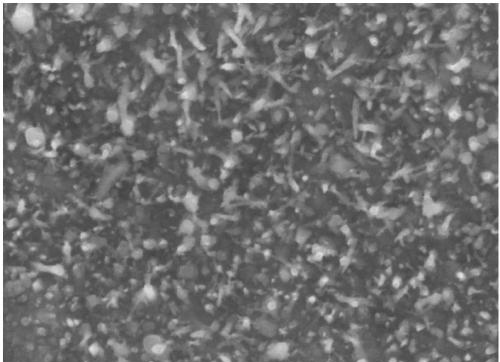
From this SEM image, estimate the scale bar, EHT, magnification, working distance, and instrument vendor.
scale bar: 1000 nm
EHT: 20 kV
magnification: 50 K X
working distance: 14.11 mm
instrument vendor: Tescan